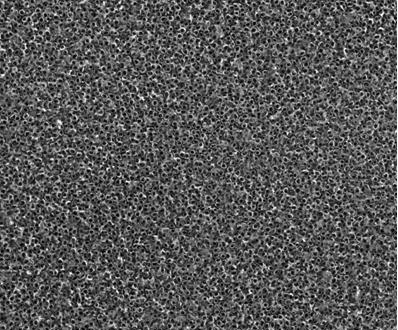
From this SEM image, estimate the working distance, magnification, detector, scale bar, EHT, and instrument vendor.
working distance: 6.6 mm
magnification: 100 K X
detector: TLD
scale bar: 500 nm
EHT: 20 kV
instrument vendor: FEI